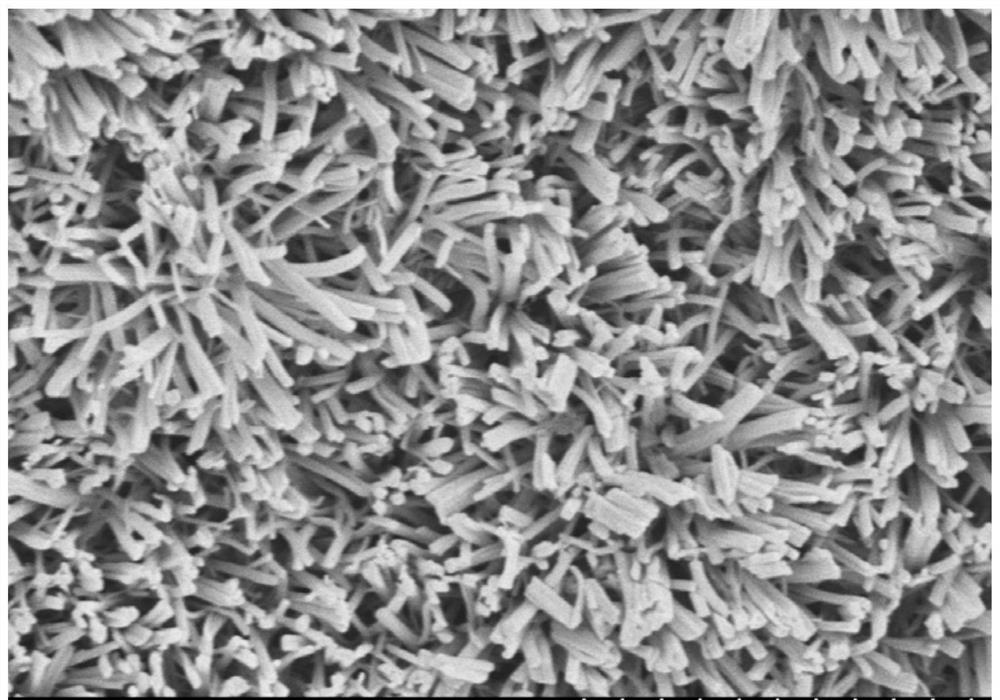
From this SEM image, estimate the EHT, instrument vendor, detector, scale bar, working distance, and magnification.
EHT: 5 kV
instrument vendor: Hitachi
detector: SE(M,LA0)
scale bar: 10000 nm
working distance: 12.8 mm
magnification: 5 K X